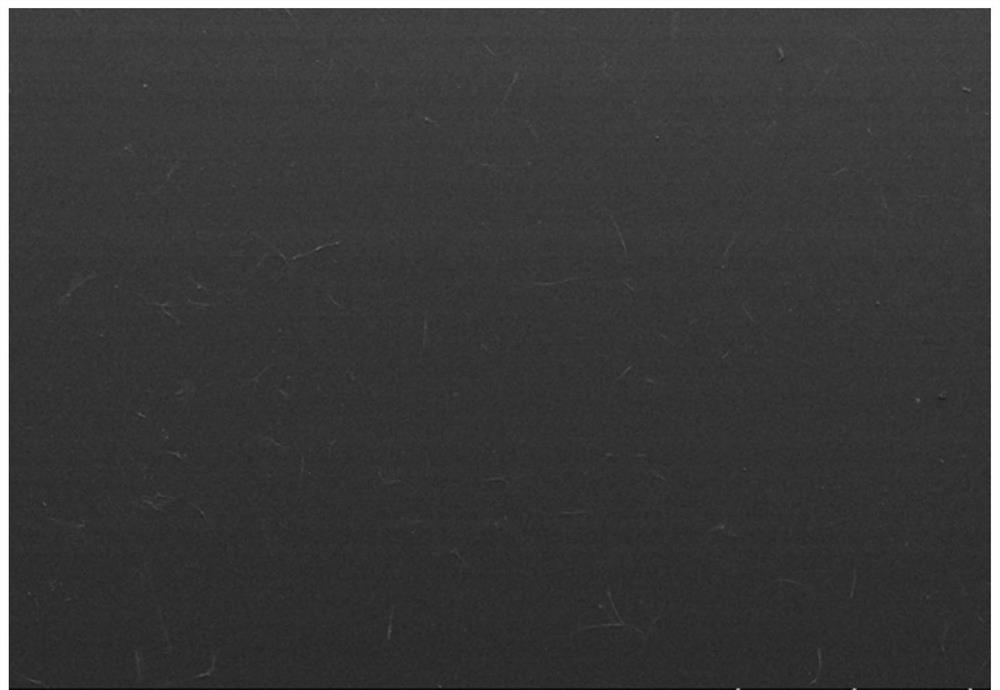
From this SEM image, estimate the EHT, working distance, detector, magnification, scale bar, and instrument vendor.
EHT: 10 kV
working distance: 10.7 mm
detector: LM(L)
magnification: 0.03 K X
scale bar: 1000000 nm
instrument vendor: Hitachi